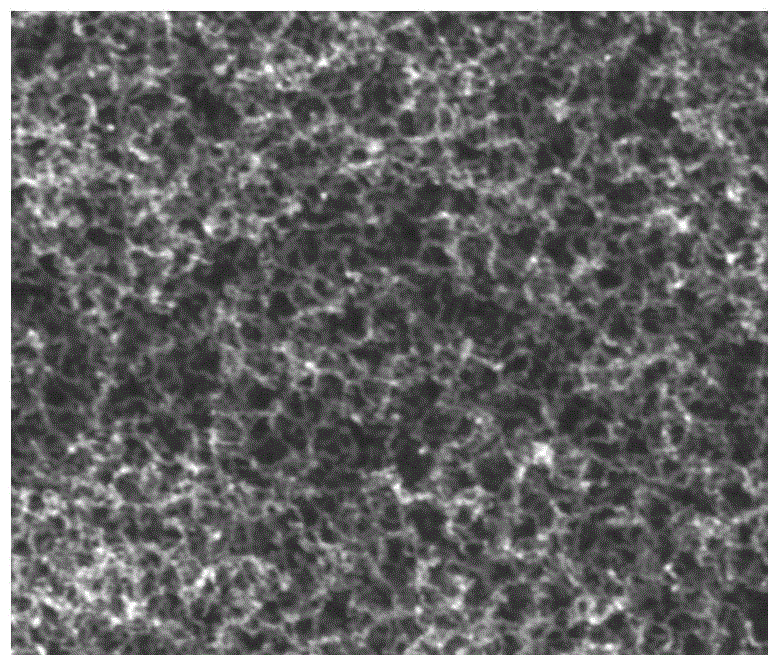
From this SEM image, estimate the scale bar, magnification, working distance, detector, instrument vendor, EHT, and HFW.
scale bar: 400 nm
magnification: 100 K X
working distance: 5.3 mm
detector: TLD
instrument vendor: FEI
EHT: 15 kV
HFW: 1.28 µm